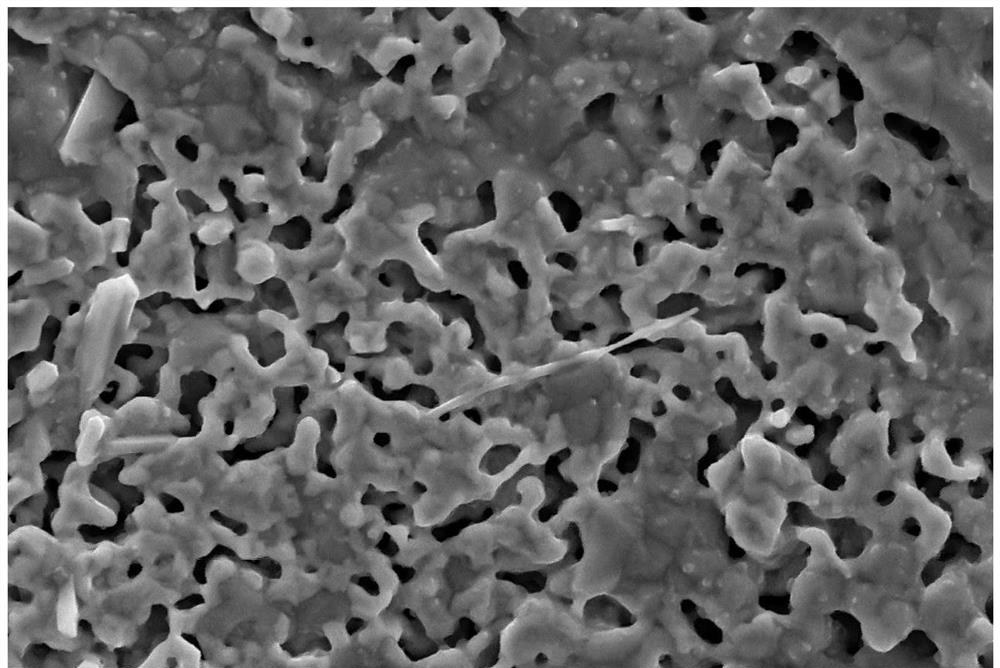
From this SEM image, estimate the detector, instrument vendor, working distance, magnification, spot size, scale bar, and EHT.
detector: SE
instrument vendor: other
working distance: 7.4 mm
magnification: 5 K X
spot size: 10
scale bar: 10000 nm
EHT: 20 kV